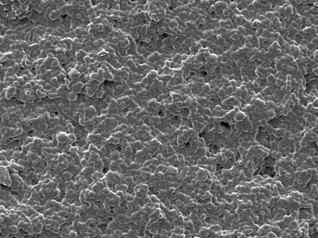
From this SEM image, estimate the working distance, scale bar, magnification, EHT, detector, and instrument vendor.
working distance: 9.9 mm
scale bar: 10000 nm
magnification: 1 K X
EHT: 5 kV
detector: LED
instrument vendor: JEOL